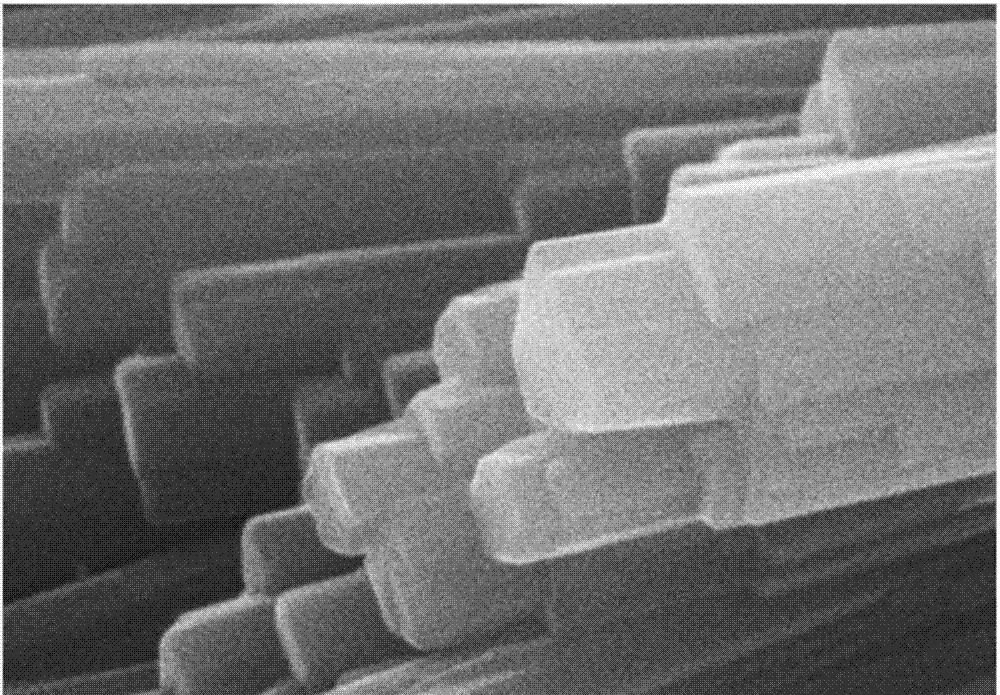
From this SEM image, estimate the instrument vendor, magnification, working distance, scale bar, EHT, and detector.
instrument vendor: Hitachi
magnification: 20 K X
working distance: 7.9 mm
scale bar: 2000 nm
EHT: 20 kV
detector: SE